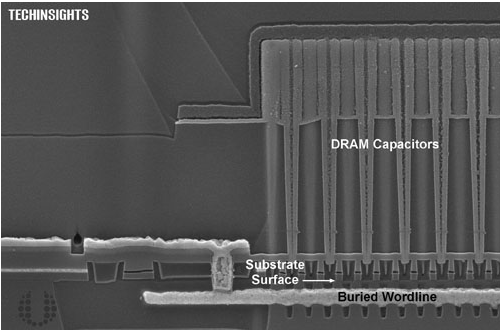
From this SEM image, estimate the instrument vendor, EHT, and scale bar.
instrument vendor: Zeiss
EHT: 5 kV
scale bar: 200 nm